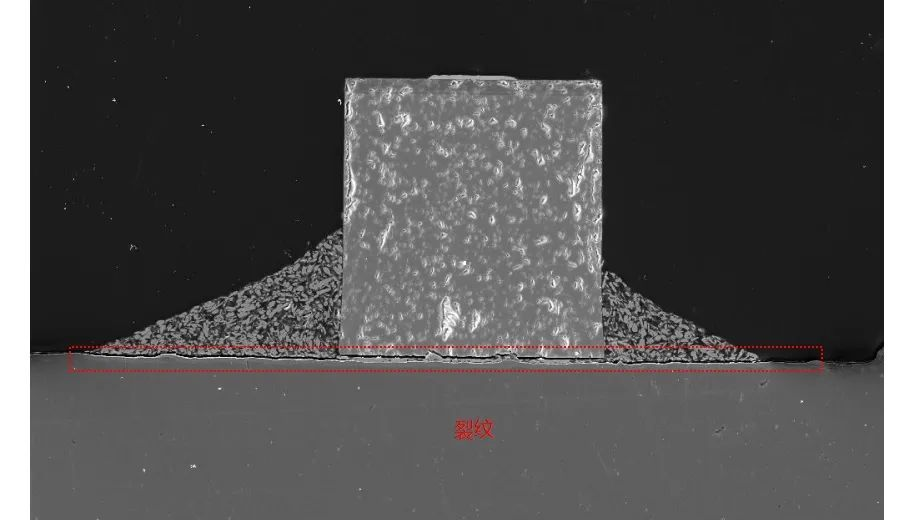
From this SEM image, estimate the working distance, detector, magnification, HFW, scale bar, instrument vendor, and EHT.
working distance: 19.87 mm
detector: SE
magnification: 0.494 K X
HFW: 560 µm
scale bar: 100000 nm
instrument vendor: Tescan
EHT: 20 kV